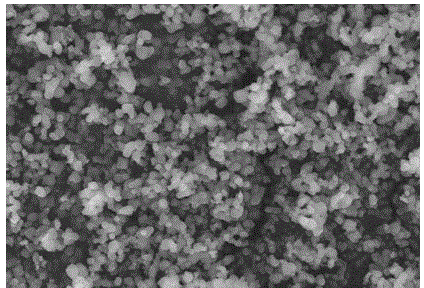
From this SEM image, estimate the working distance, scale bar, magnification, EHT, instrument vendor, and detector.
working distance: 7.9 mm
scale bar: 10000 nm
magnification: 5 K X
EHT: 20 kV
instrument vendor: Hitachi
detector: SE(M)